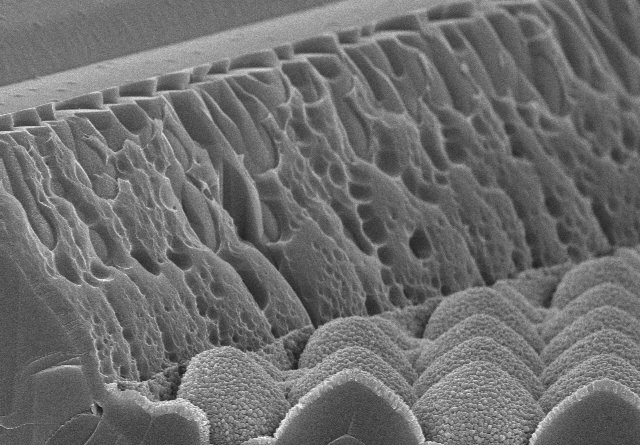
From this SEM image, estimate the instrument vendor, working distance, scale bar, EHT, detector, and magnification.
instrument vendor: Hitachi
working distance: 8.3 mm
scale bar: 5000 nm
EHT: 10 kV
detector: SE(U)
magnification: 10 K X